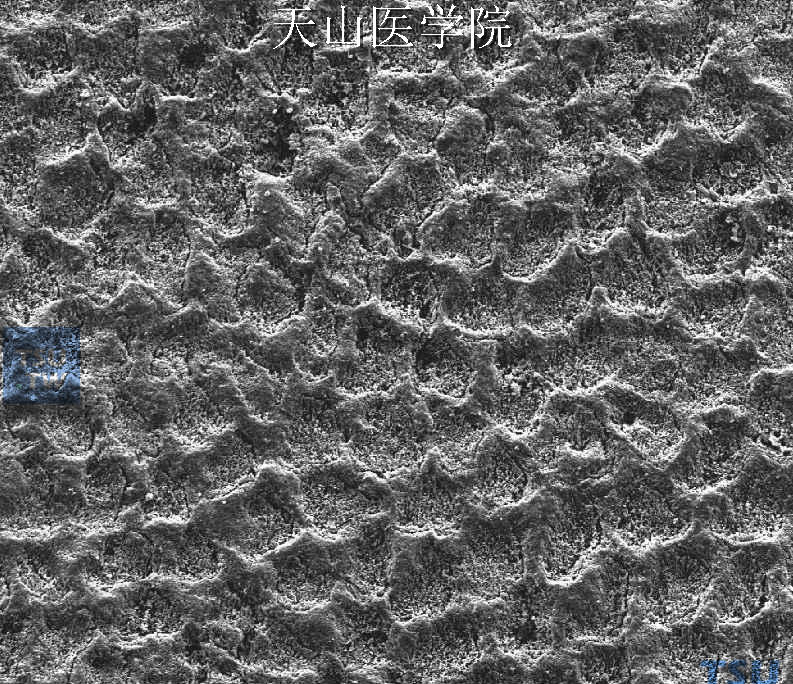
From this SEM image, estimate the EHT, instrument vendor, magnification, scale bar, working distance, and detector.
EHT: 5 kV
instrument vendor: FEI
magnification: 5 K X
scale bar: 20000 nm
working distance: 12.1 mm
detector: SE